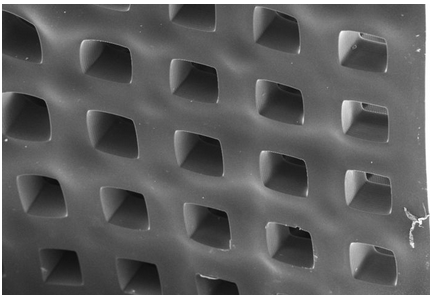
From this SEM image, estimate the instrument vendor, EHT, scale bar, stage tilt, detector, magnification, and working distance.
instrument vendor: Zeiss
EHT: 10 kV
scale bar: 20000 nm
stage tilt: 45°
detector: SE2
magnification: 0.5 K X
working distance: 9.1 mm